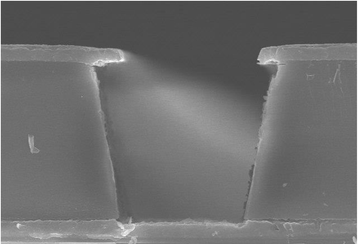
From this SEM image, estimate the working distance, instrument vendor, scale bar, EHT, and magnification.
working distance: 12.9 mm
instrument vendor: Hitachi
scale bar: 10000 nm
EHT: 15 kV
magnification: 4.5 K X